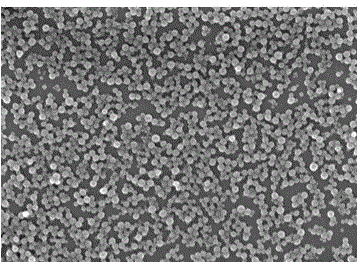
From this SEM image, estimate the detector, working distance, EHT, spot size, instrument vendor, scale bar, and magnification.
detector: TLD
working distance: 6.4 mm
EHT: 5 kV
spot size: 4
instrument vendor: FEI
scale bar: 500 nm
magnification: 40 K X